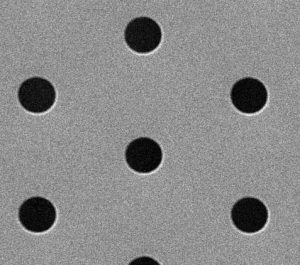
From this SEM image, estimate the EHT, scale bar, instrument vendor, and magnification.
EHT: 25 kV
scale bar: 5000 nm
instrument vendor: JEOL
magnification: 5 K X